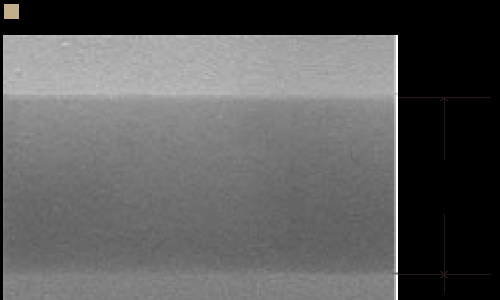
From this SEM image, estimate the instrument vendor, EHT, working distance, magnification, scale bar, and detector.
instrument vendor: Hitachi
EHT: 10 kV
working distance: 4.7 mm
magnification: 100 K X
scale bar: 500 nm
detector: SE(U)